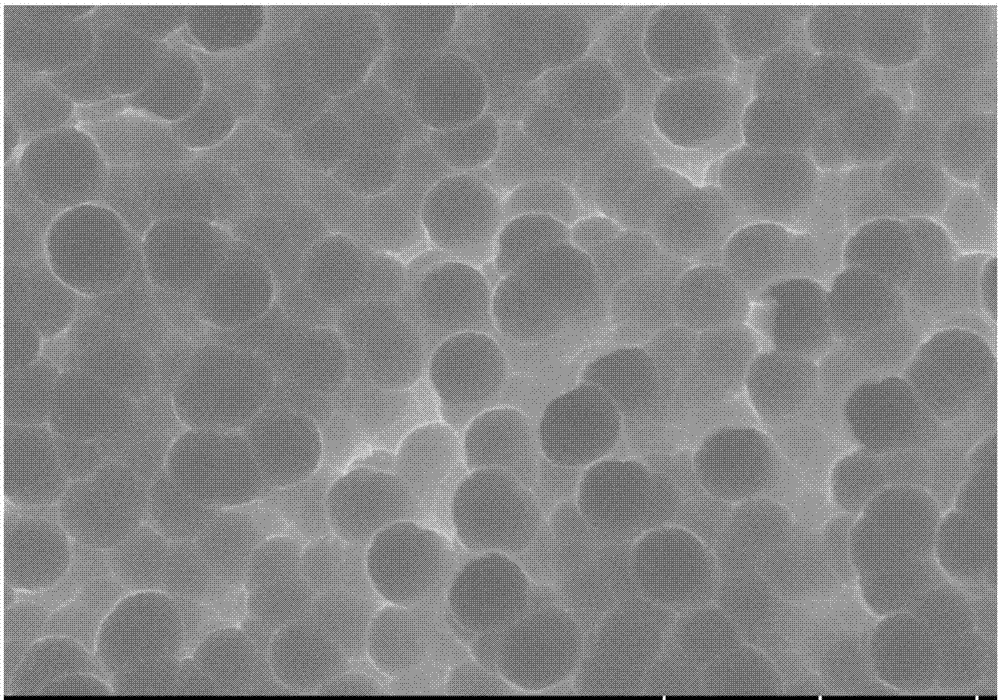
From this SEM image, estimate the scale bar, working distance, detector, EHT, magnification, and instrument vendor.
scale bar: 2000 nm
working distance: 8 mm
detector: SE(UL)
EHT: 10 kV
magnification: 20 K X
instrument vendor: Hitachi